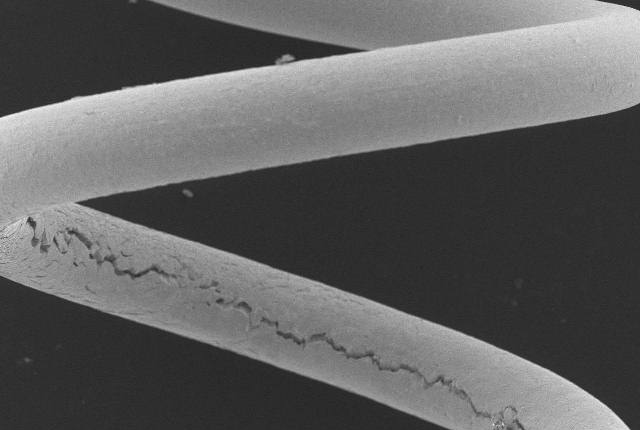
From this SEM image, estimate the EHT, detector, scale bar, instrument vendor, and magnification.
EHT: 15 kV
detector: SEI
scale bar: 200000 nm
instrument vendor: JEOL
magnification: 0.085 K X